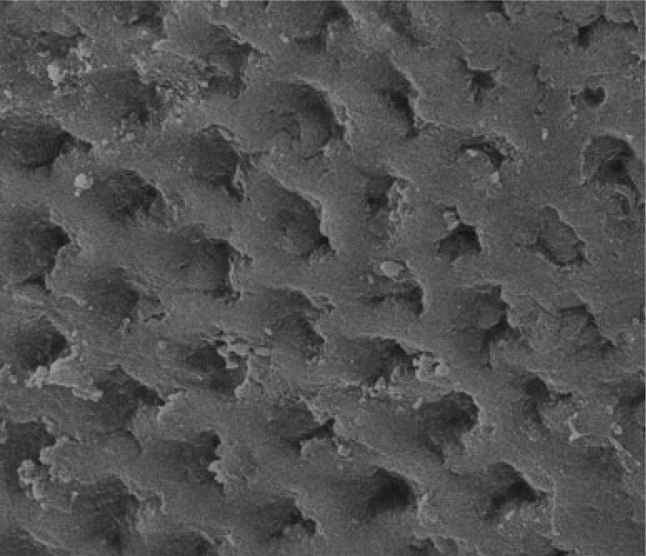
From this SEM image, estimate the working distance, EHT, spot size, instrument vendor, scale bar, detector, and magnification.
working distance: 10.6 mm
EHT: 20 kV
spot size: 2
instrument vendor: FEI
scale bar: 20000 nm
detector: ETD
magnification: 6 K X